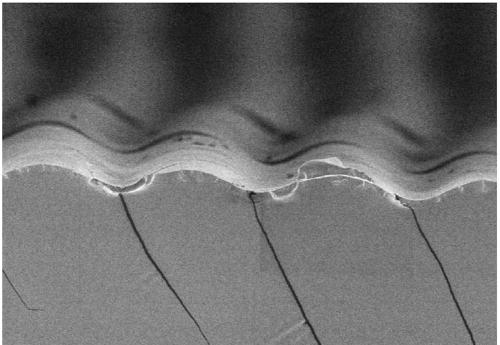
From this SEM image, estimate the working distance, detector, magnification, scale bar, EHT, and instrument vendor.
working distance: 11.2 mm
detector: SE(M)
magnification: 0.5 K X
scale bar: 100000 nm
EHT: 3 kV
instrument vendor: Hitachi